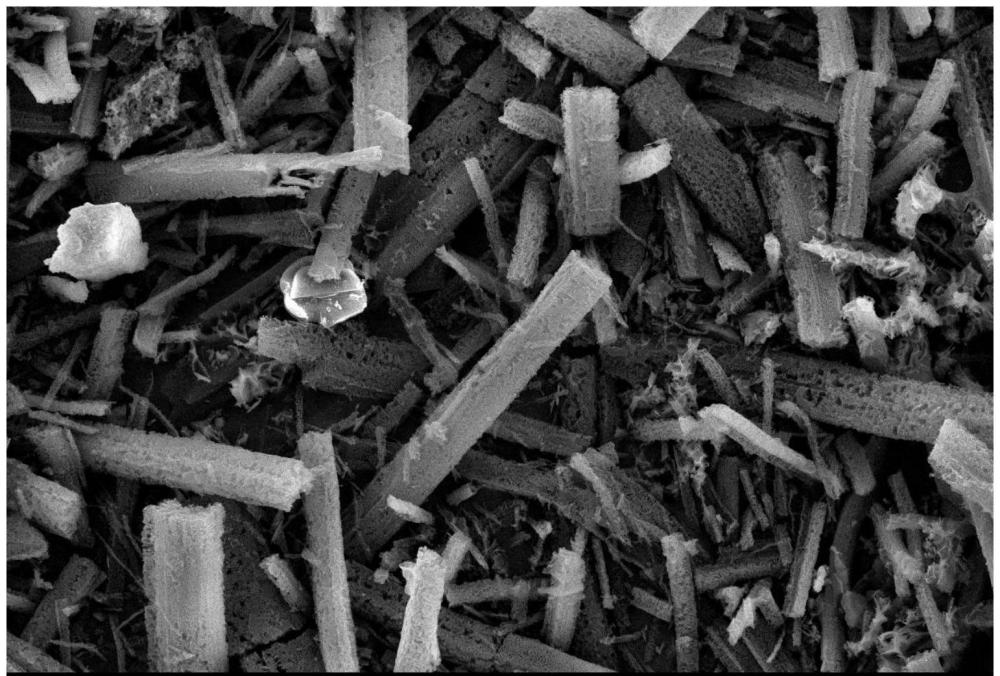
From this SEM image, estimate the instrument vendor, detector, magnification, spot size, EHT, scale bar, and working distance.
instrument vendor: other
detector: SE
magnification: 1 K X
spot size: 8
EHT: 15 kV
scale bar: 50000 nm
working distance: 10 mm